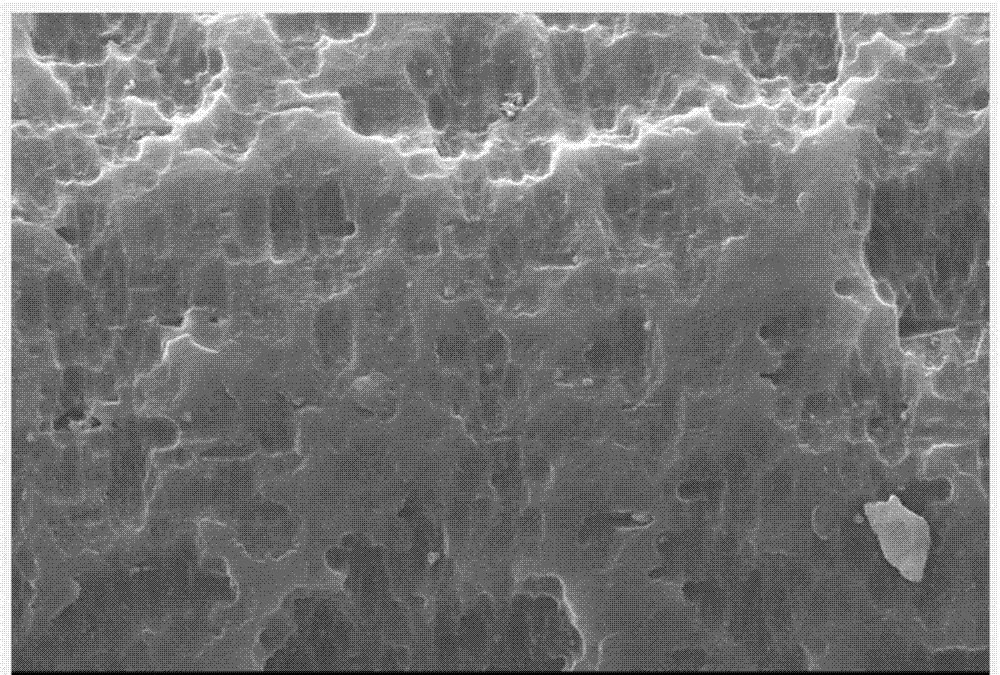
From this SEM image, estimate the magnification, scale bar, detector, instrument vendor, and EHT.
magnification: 3 K X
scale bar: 5000 nm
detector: SEI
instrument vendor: JEOL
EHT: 15 kV